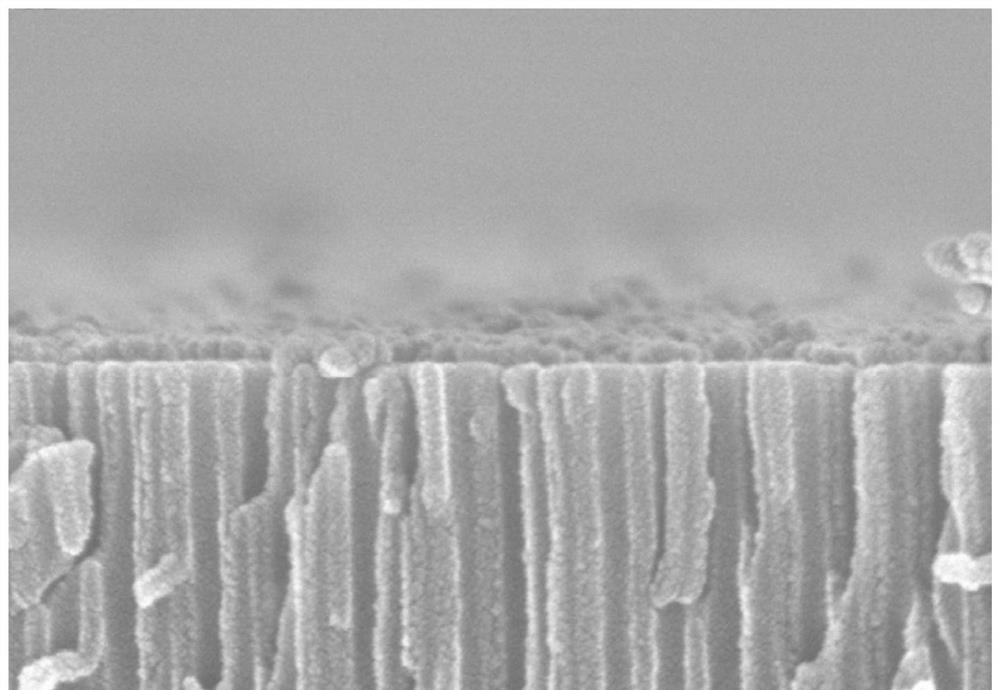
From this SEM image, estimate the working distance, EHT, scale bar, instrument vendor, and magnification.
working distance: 11.2 mm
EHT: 15 kV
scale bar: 500 nm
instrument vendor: Hitachi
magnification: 100 K X